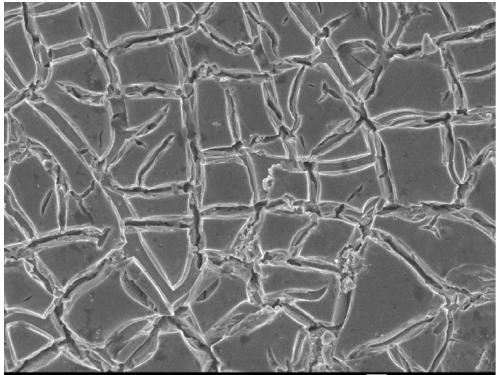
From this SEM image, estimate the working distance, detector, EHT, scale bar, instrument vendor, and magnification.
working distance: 7.3 mm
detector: SEI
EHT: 5 kV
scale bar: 1000 nm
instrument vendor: JEOL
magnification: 5 K X